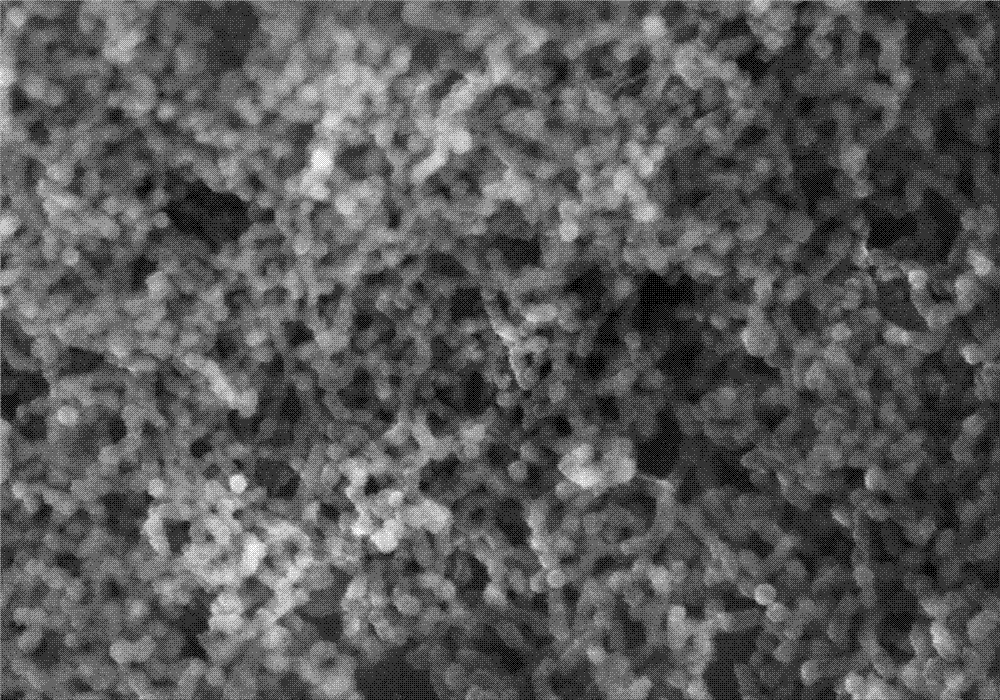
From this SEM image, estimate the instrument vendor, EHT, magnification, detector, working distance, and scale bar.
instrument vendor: Hitachi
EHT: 10 kV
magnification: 100 K X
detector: SE(U)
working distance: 4.4 mm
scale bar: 500 nm